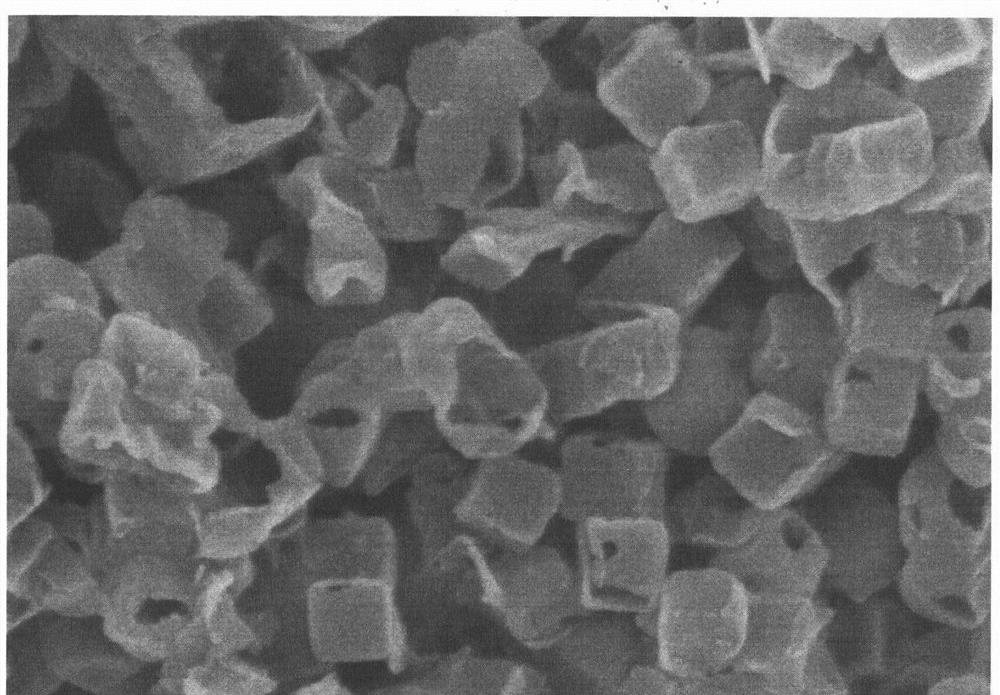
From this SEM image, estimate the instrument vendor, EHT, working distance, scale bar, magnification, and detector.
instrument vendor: Hitachi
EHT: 5 kV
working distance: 9.7 mm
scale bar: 1000 nm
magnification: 40 K X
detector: SE(M)